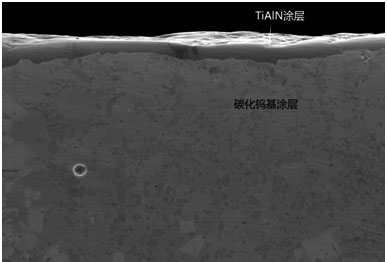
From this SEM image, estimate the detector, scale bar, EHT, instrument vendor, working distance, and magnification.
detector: ETD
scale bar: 30000 nm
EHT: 15 kV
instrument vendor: FEI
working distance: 9.4 mm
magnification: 2 K X